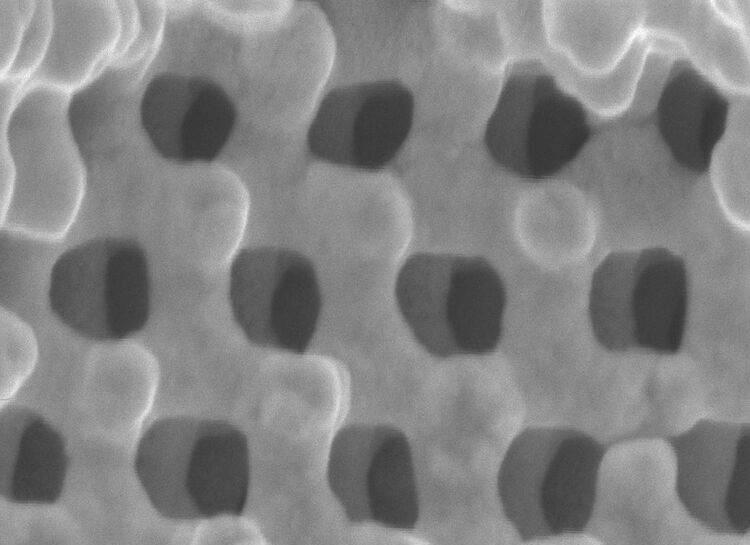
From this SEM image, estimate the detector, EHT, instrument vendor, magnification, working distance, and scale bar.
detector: SEI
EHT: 4 kV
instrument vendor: JEOL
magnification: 95 K X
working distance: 8 mm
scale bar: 100 nm